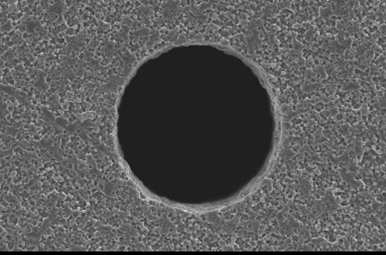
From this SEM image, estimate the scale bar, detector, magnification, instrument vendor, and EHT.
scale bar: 50000 nm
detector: SEI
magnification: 0.4 K X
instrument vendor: JEOL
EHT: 20 kV